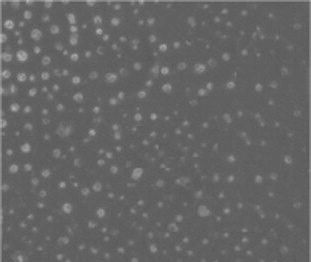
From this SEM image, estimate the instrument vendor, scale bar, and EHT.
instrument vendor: FEI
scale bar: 500 nm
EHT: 20 kV